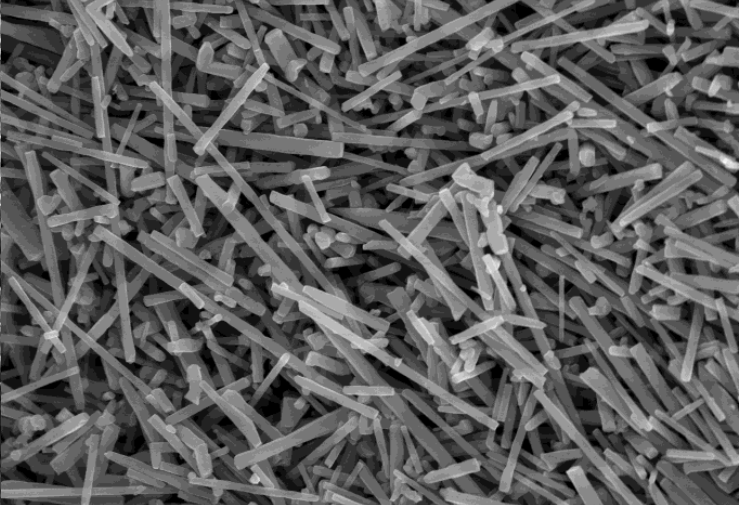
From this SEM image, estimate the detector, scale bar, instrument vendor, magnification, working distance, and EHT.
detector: SE(UL)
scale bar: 10000 nm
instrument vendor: Hitachi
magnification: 10 K X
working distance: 9.8 mm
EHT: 15 kV